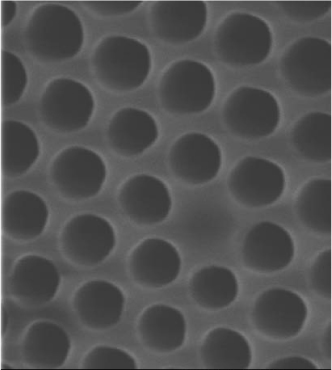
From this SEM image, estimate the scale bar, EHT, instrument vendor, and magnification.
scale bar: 2330 nm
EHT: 15 kV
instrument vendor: JEOL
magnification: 12.9 K X